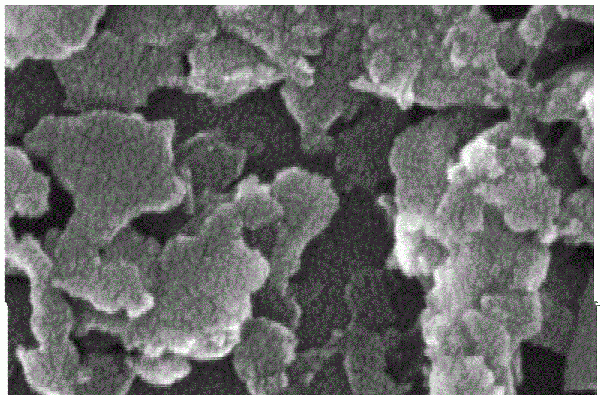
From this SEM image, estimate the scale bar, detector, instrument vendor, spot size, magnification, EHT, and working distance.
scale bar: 300 nm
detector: TLD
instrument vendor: FEI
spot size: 3.5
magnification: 200 K X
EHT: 15 kV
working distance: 6.4 mm